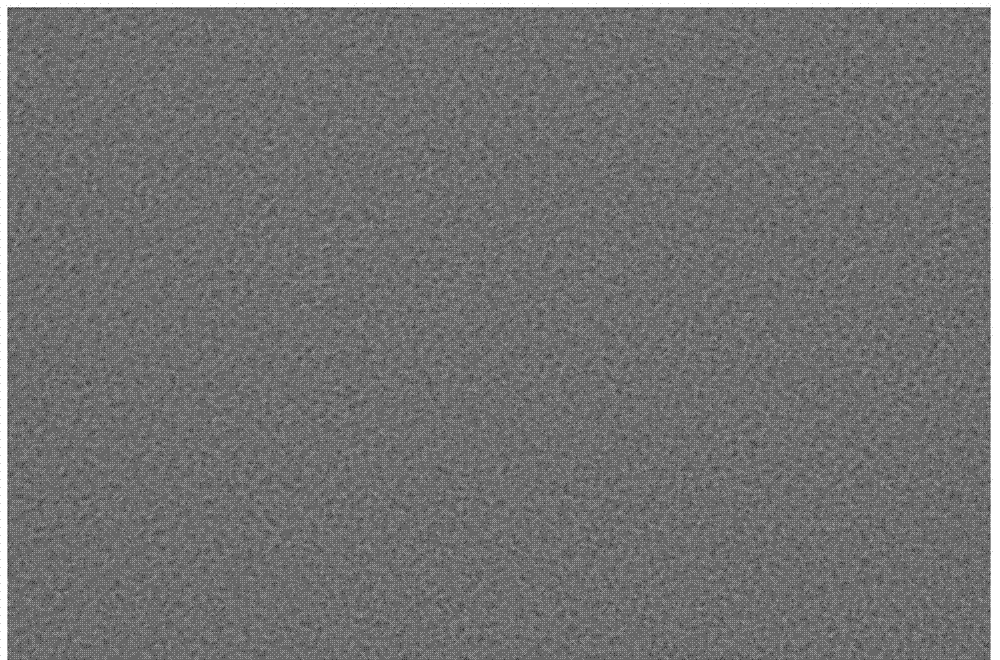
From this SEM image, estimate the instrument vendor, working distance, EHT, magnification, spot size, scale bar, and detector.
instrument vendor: FEI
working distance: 2.8 mm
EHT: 0.8 kV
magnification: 80 K X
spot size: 3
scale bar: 2000 nm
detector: CBS